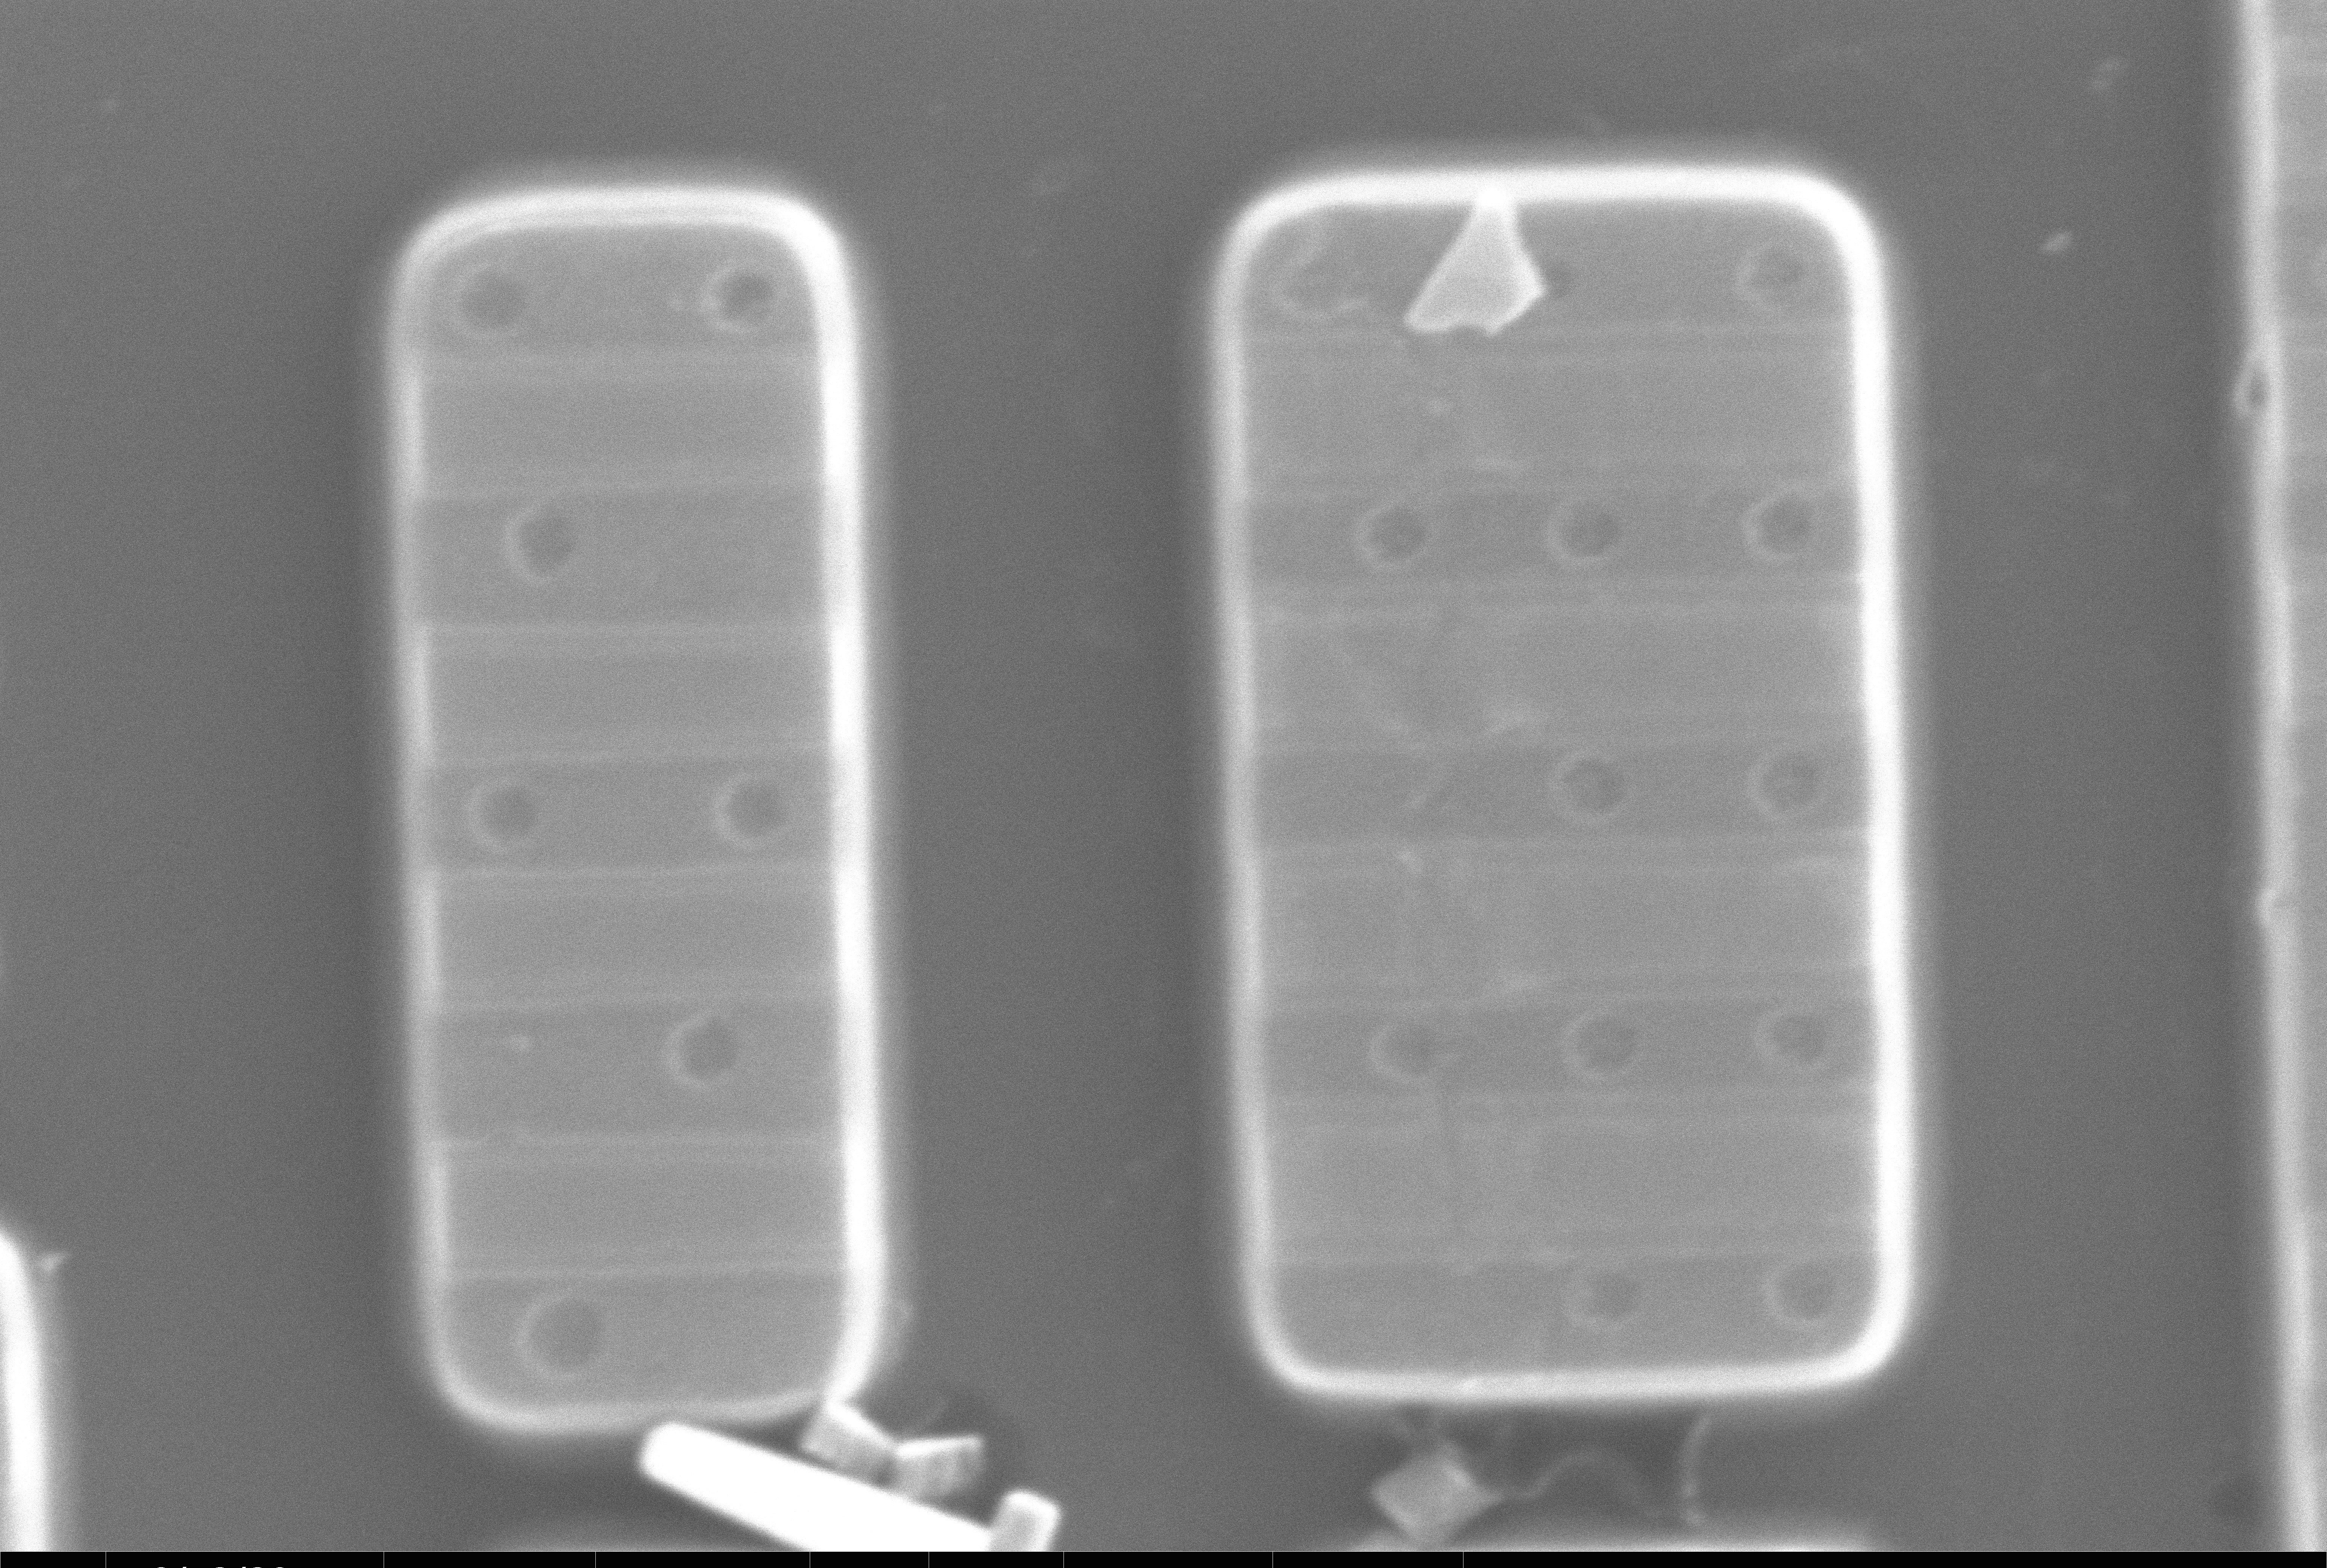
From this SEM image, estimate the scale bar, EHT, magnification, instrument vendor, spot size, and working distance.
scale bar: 1000 nm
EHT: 20 kV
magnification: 50 K X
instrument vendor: FEI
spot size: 6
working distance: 10.6 mm